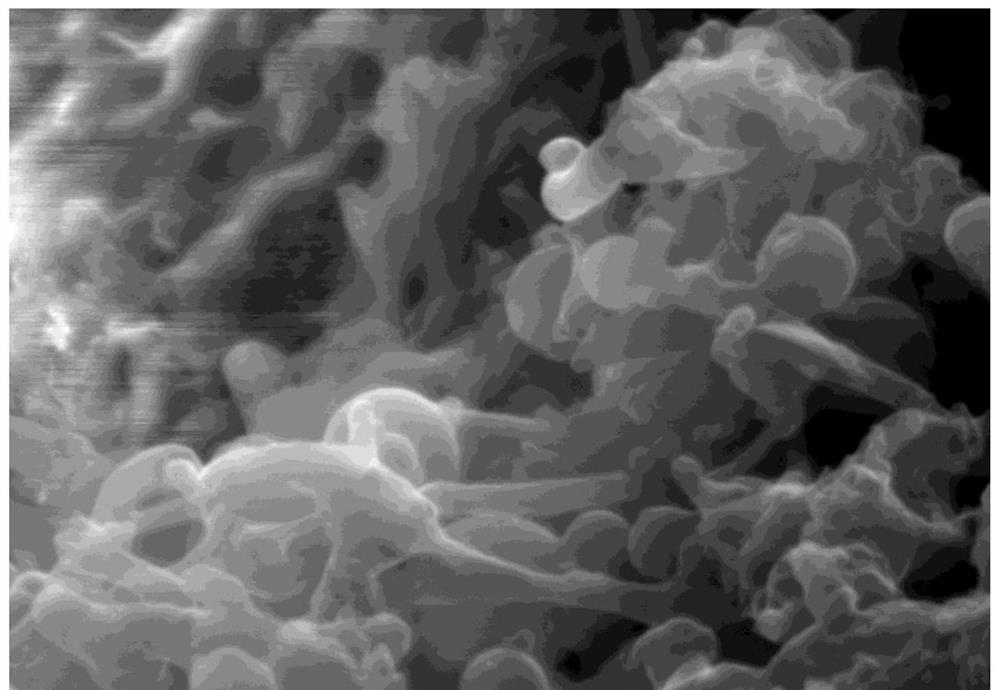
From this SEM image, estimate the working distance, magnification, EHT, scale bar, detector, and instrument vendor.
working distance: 13.9 mm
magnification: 50 K X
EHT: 20 kV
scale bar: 1000 nm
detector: SE(L)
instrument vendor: Hitachi